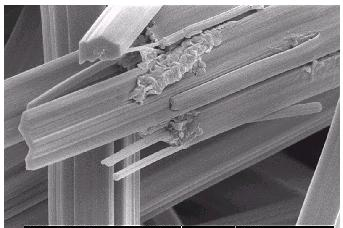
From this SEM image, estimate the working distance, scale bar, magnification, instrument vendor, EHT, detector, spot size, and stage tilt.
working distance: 4.7 mm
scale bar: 2000 nm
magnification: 10 K X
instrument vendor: FEI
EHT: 5 kV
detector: SE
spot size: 2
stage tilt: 0°